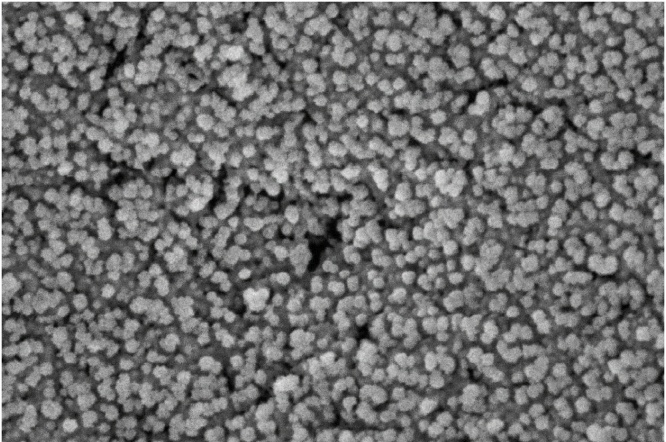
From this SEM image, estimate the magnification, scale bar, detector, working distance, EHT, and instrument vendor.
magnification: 106.93 K X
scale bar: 100 nm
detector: InLens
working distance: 3.1 mm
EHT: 4 kV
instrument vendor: Zeiss